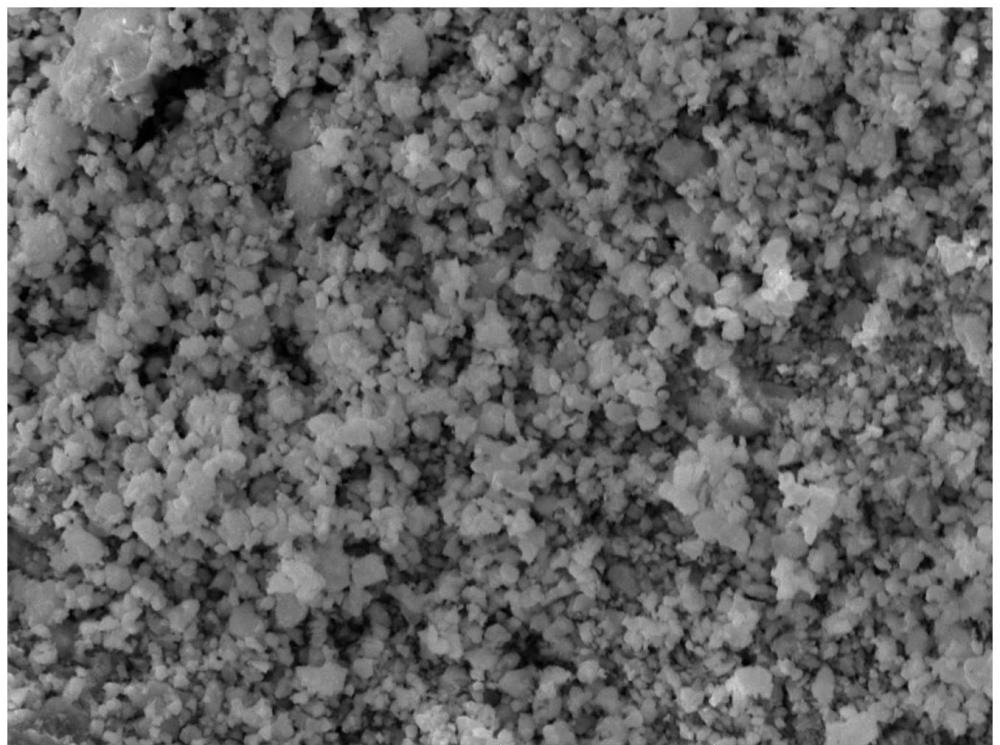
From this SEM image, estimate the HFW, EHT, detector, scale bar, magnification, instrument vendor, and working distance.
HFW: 46.1 µm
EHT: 30 kV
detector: SE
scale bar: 10000 nm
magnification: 6 K X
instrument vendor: Tescan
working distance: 10 mm